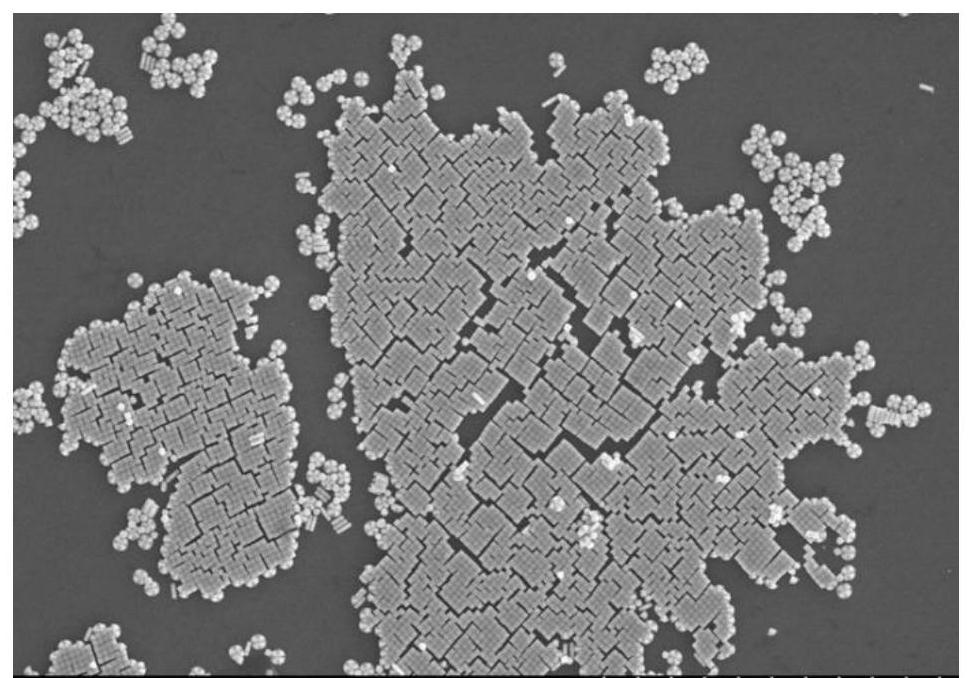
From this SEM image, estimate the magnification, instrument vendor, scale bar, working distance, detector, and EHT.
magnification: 15 K X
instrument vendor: Hitachi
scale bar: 3000 nm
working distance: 11.8 mm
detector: SE(M)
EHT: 5 kV